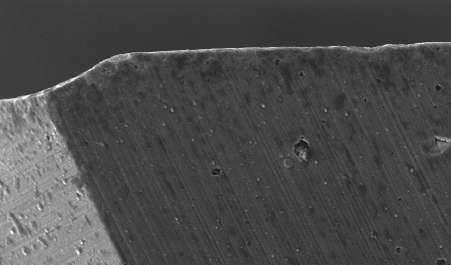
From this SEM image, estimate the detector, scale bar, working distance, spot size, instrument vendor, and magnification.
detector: SE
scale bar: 100000 nm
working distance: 12.4 mm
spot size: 4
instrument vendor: FEI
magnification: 0.35 K X